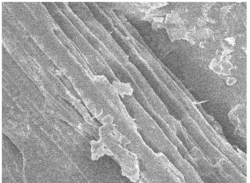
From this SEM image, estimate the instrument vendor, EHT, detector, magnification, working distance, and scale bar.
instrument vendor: JEOL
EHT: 10 kV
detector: SEI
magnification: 1 K X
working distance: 8 mm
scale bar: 10000 nm